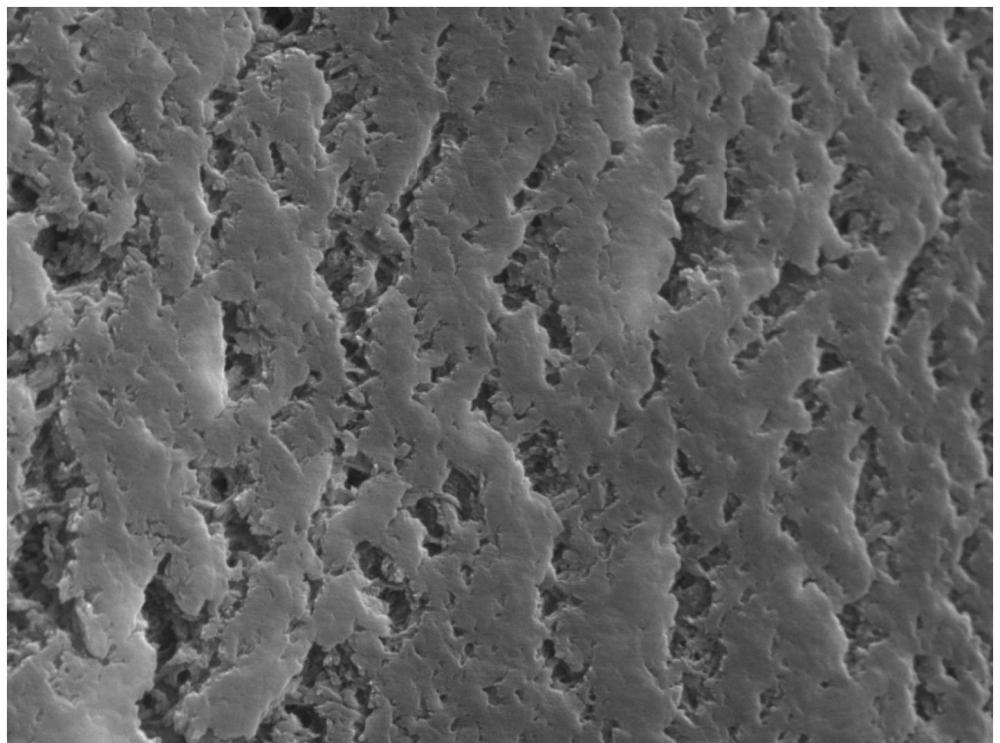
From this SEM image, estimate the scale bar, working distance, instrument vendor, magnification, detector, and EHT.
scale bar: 5000 nm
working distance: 8.56 mm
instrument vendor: Tescan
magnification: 6.01 K X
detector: SE + BSE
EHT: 20 kV